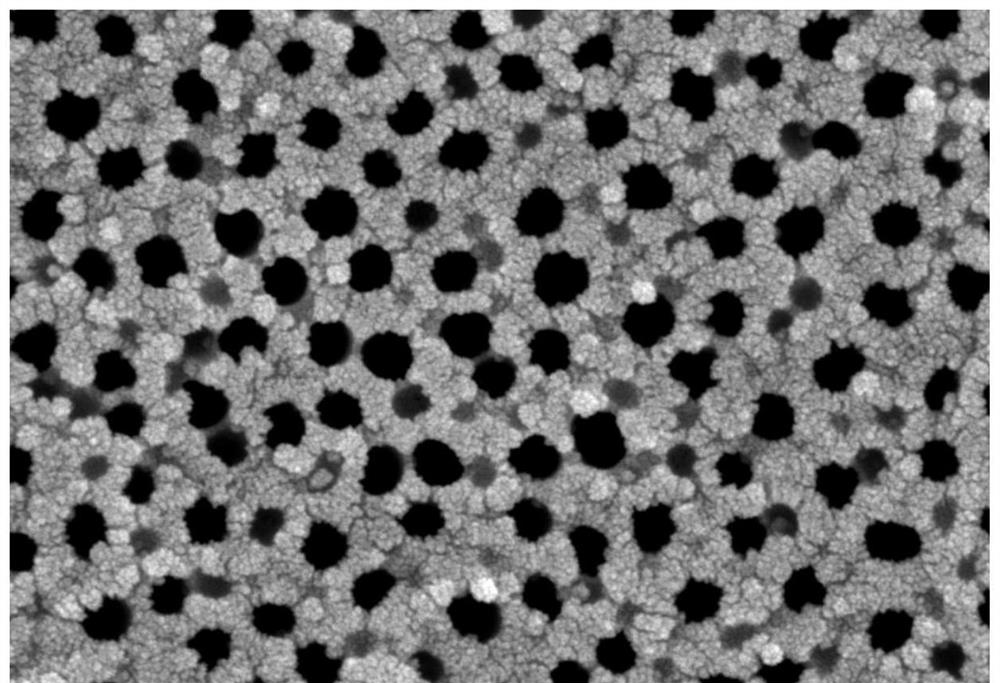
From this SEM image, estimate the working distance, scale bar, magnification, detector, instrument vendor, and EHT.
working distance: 4.4 mm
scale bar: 100 nm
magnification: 100 K X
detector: InLens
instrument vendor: Zeiss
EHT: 3 kV